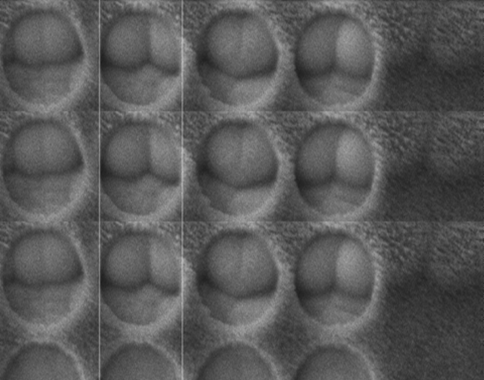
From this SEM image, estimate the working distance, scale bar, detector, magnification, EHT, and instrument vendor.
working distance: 13.8 mm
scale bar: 1000 nm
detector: LEI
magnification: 16 K X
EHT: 5 kV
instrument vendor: JEOL